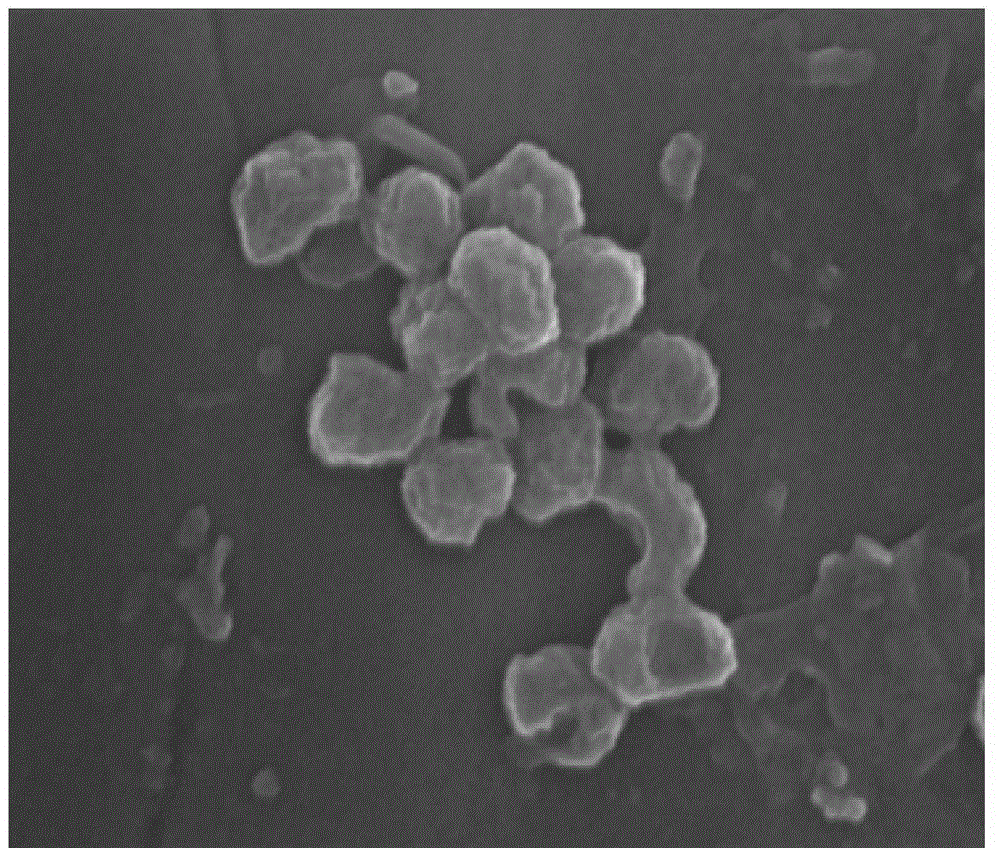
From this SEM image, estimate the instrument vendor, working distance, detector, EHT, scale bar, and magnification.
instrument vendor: FEI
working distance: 5.8 mm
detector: TLD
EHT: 10 kV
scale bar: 200 nm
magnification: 200 K X